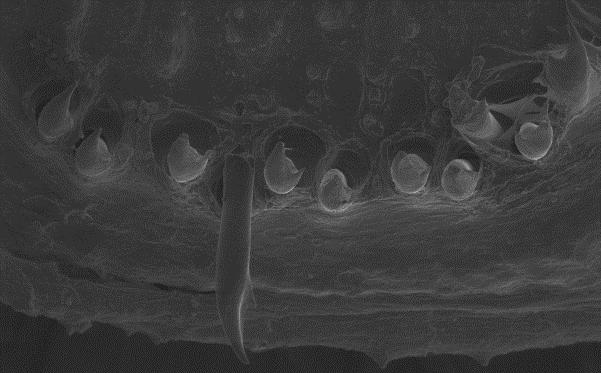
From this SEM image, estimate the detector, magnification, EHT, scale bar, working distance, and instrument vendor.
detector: SE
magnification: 0.125 K X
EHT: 15 kV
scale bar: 100000 nm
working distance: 9 mm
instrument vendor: other